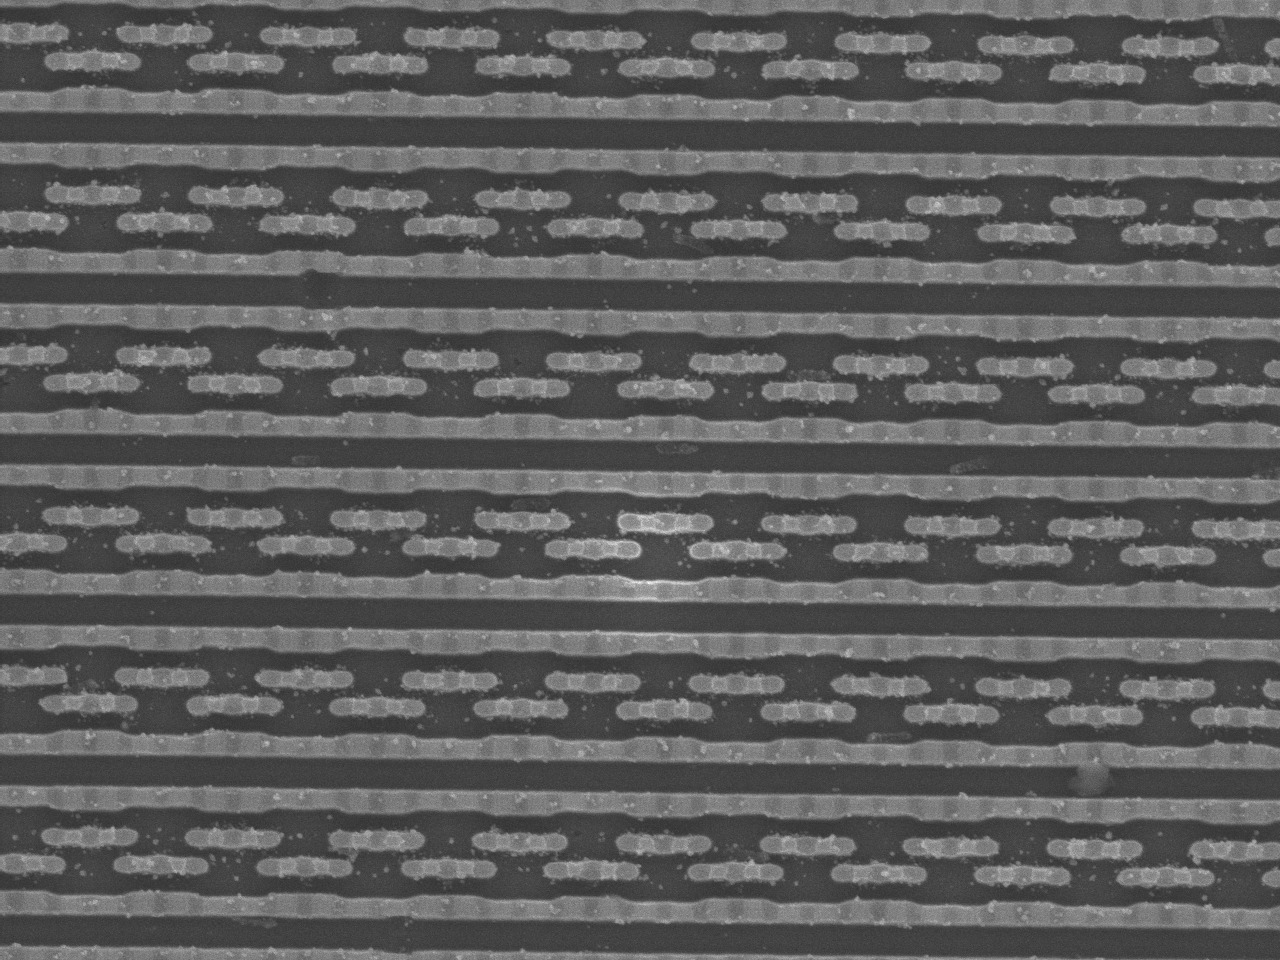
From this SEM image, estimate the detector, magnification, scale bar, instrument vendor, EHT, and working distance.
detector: SEI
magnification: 10 K X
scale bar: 1000 nm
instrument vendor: JEOL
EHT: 5 kV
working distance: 15.2 mm